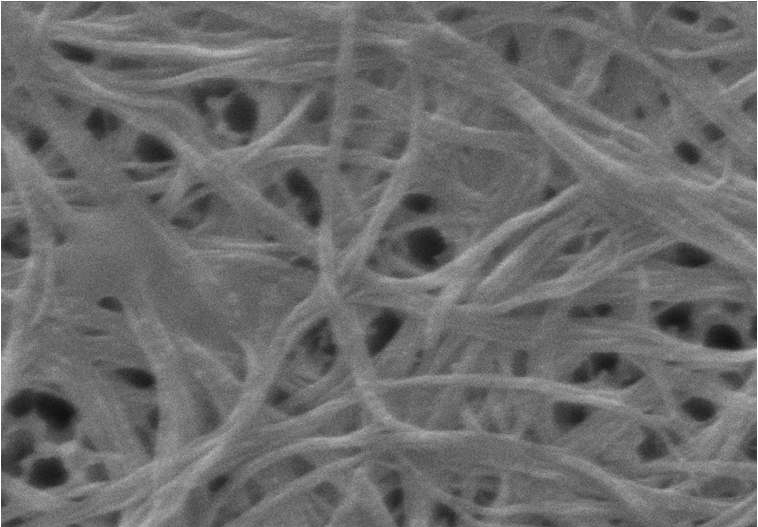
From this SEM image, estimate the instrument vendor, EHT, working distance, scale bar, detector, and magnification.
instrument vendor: Zeiss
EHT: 20 kV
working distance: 11 mm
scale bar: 100 nm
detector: SE1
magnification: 80 K X